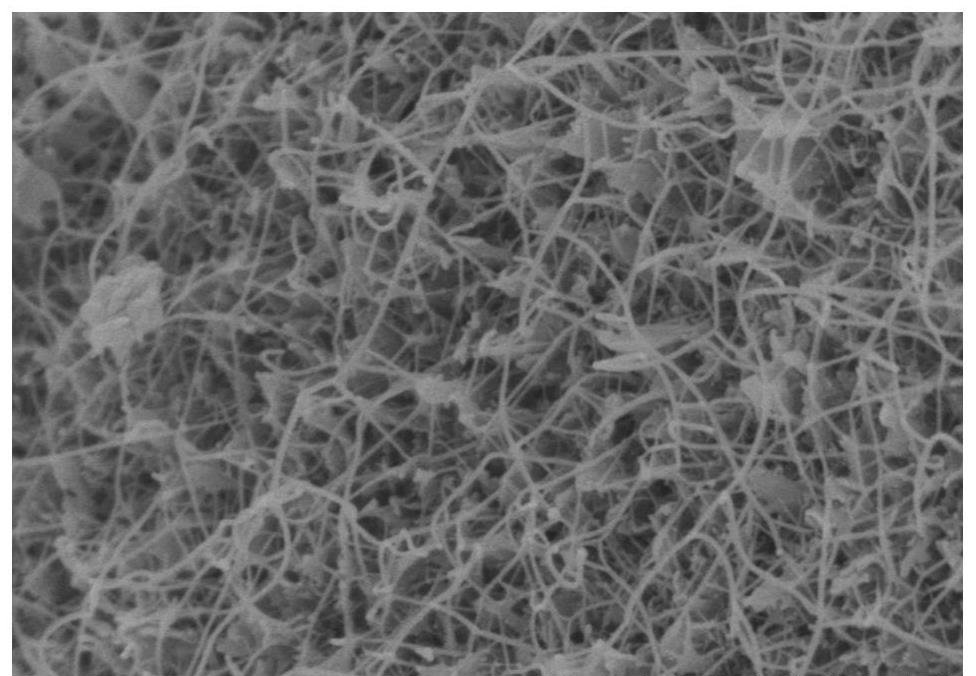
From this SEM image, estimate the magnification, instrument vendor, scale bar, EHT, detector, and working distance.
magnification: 50 K X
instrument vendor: Hitachi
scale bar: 1000 nm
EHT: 3 kV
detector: SE(M)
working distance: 9.3 mm